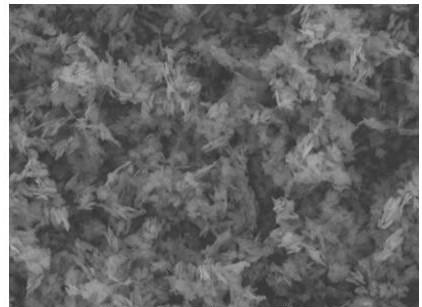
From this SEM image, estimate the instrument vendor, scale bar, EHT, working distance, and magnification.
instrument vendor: Tescan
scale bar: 5000 nm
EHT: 20 kV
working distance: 15.01 mm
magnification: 5 K X